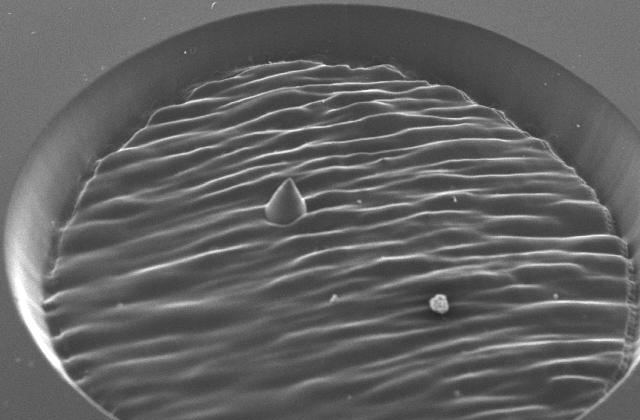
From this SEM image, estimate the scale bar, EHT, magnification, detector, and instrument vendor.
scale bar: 200000 nm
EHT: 20 kV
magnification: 0.06 K X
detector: SEI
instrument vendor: JEOL